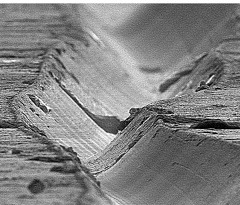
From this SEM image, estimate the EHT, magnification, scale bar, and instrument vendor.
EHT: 20 kV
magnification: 0.48 K X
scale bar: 20000 nm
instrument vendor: JEOL